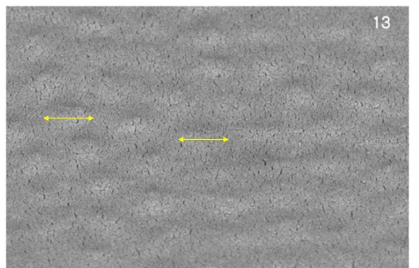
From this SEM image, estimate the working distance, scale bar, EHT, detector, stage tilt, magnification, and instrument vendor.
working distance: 4 mm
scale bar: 500 nm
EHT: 2 kV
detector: TLD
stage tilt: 52°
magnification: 100 K X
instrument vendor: FEI